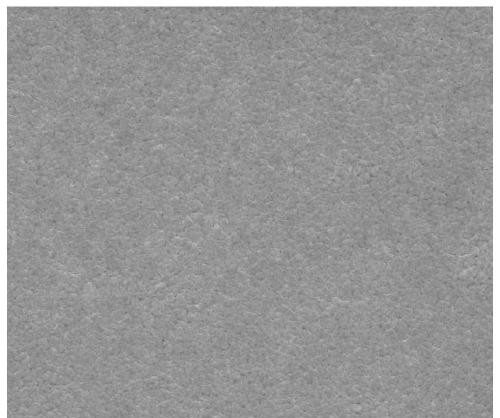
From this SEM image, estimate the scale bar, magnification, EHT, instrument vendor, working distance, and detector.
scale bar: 2000 nm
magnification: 50 K X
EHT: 20 kV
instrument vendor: FEI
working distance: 10.6 mm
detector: ETD